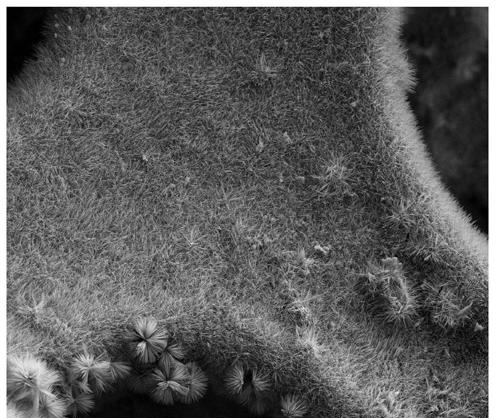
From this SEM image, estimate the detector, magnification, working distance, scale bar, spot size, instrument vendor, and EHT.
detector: ETD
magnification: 2 K X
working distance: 4.8 mm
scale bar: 40000 nm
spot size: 3.5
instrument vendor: FEI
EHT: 15 kV